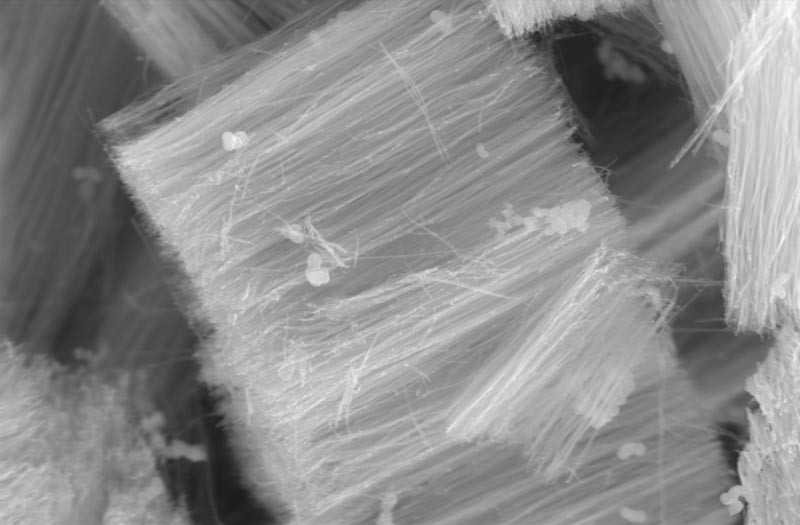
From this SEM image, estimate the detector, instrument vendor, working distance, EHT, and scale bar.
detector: SE1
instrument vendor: Zeiss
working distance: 5 mm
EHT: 15 kV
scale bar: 2000 nm